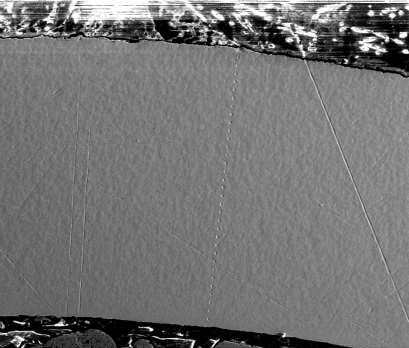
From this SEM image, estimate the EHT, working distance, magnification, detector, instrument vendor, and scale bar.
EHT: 20 kV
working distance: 10 mm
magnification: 0.15 K X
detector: ETD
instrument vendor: FEI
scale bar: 400000 nm